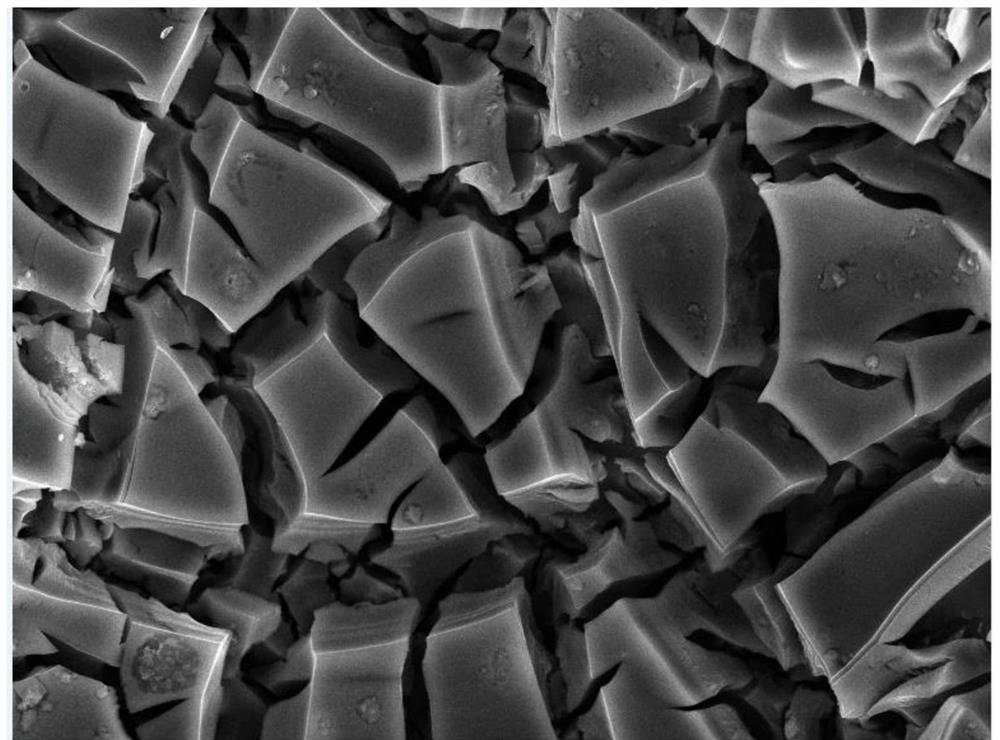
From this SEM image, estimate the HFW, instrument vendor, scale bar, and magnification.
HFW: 41.4 µm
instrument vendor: Tescan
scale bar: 10000 nm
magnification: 5 K X